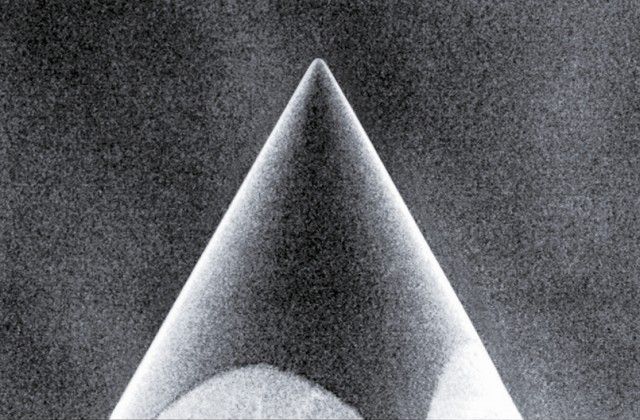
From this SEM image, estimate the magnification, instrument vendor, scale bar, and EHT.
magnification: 0.35 K X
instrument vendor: JEOL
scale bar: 50000 nm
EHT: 20 kV